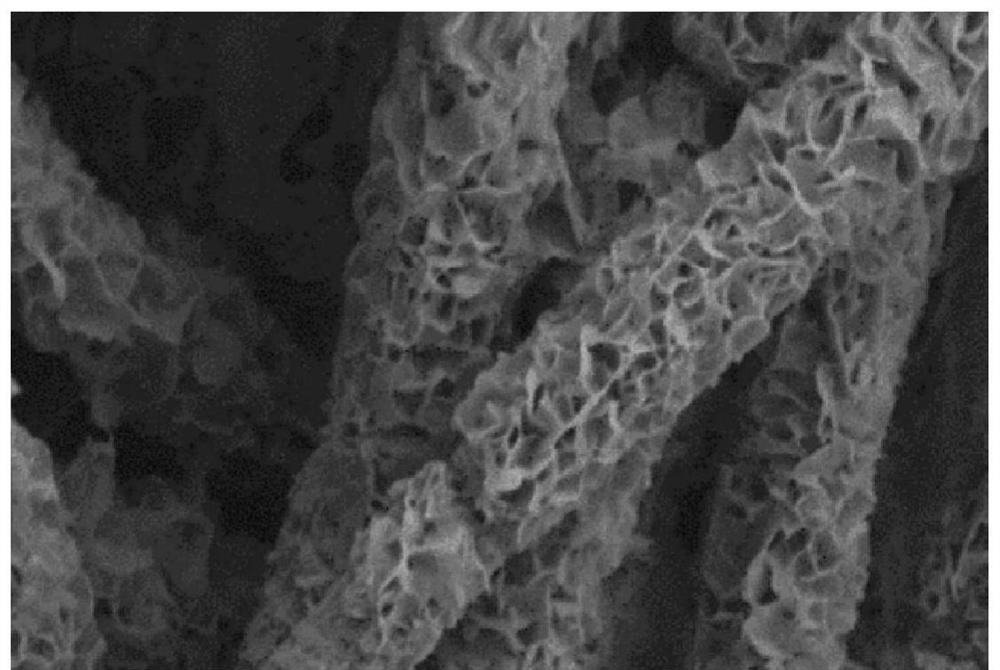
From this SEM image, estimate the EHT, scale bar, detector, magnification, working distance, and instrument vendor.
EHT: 2 kV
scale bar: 200 nm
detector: SE2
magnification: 20 K X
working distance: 6.3 mm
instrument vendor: Zeiss